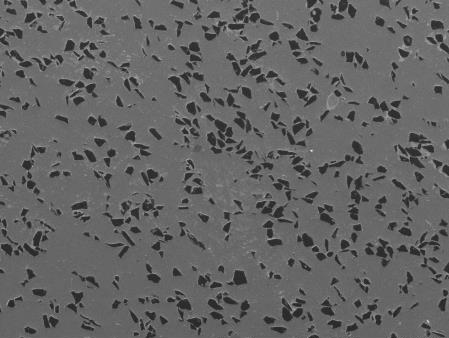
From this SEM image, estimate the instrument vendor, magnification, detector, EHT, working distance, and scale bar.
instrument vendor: JEOL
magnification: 0.075 K X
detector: LED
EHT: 15 kV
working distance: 10 mm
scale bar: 100000 nm